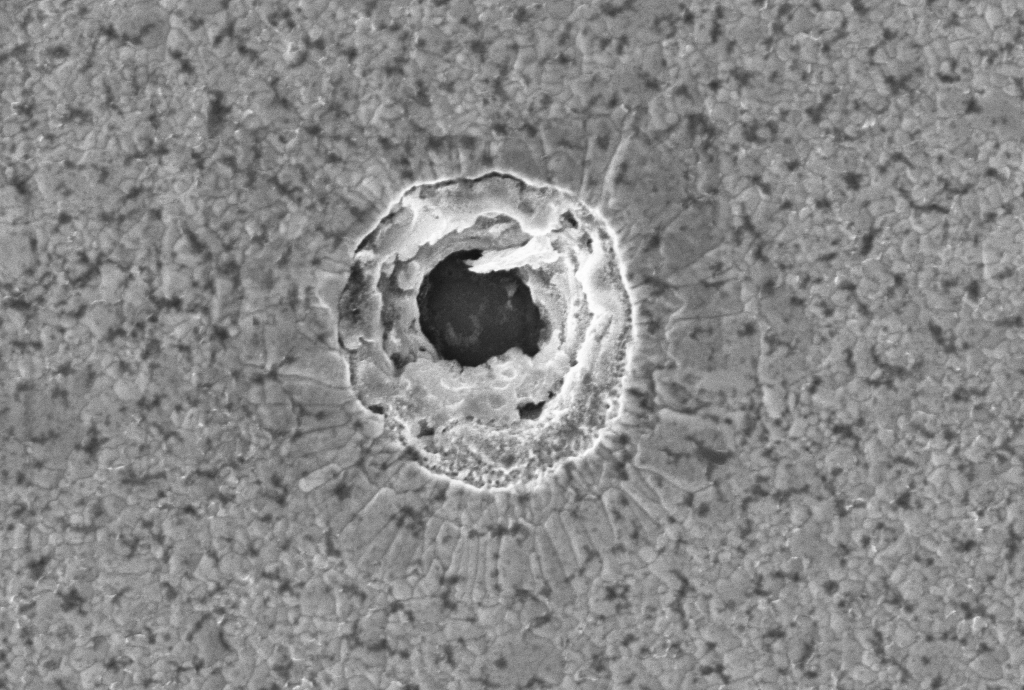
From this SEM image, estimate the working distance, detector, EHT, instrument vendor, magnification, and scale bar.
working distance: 9.4 mm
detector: InLens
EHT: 5 kV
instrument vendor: Zeiss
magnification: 15 K X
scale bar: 1000 nm